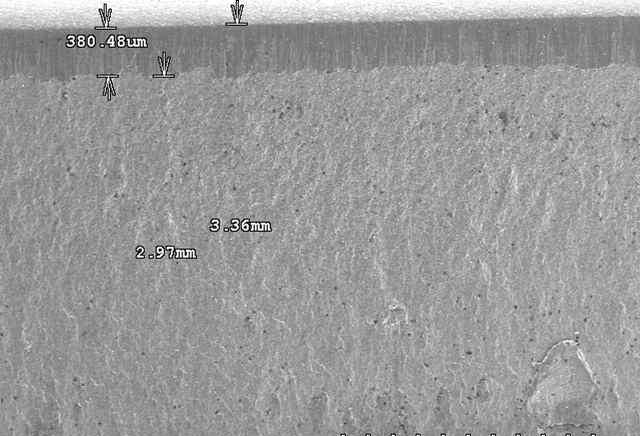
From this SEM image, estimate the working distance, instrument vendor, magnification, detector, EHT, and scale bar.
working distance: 33.7 mm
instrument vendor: JEOL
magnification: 0.025 K X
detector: SE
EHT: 15 kV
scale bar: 2000000 nm